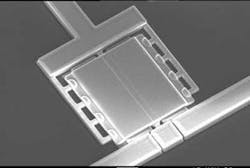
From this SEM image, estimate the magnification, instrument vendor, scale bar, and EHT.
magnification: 1 K X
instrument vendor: other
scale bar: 10000 nm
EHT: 10 kV